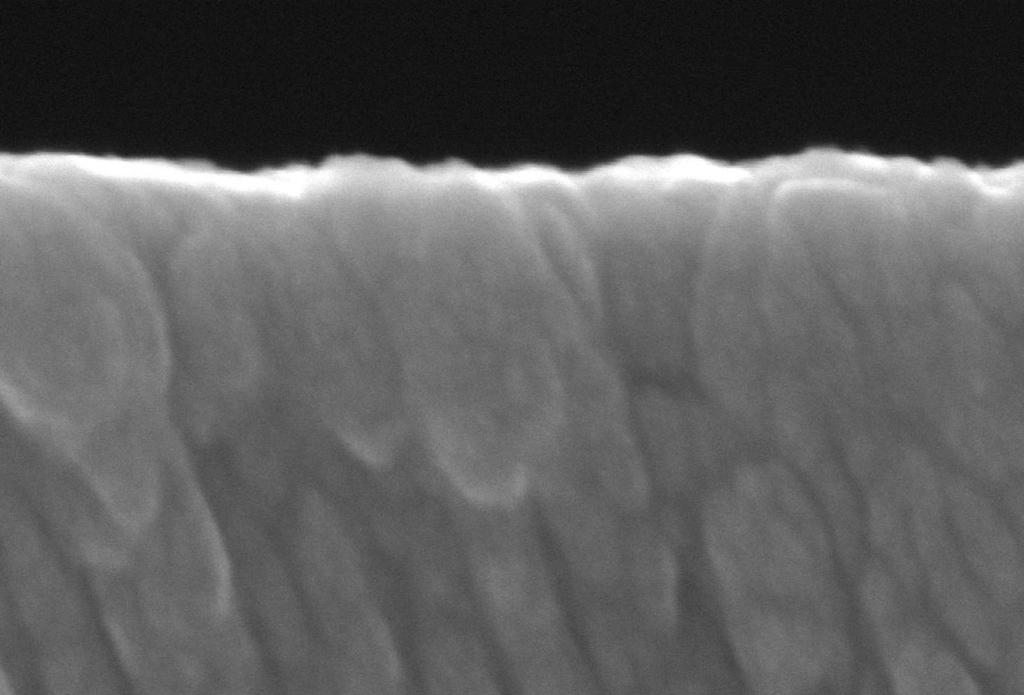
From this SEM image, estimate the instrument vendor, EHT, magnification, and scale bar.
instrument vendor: JEOL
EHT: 10 kV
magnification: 250 K X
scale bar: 100 nm